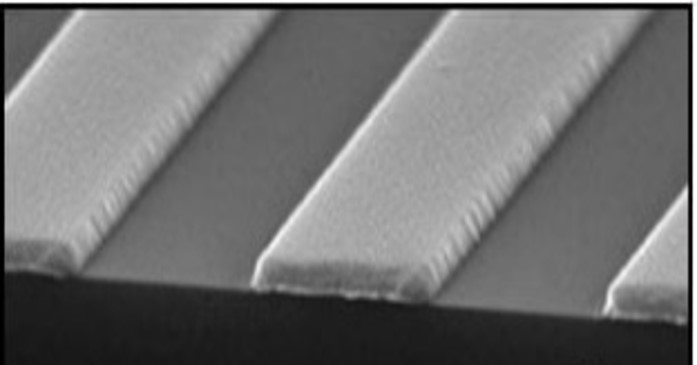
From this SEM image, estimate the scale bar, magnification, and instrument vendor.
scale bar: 1000 nm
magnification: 75 K X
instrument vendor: Zeiss